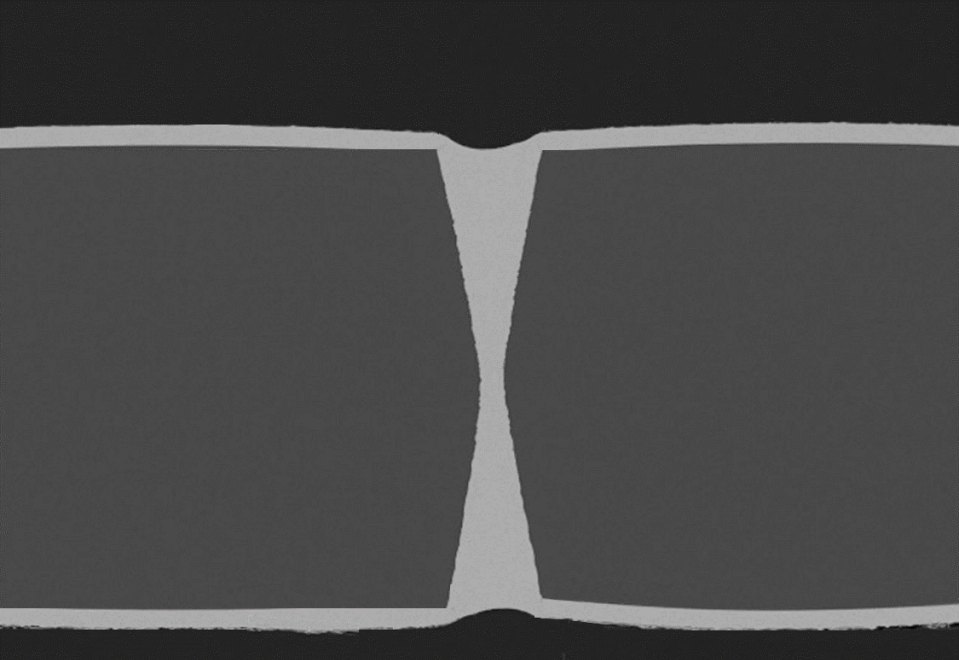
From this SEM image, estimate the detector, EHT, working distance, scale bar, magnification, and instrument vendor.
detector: BSE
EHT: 15 kV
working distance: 6.4 mm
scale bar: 200000 nm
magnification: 0.2 K X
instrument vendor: Hitachi